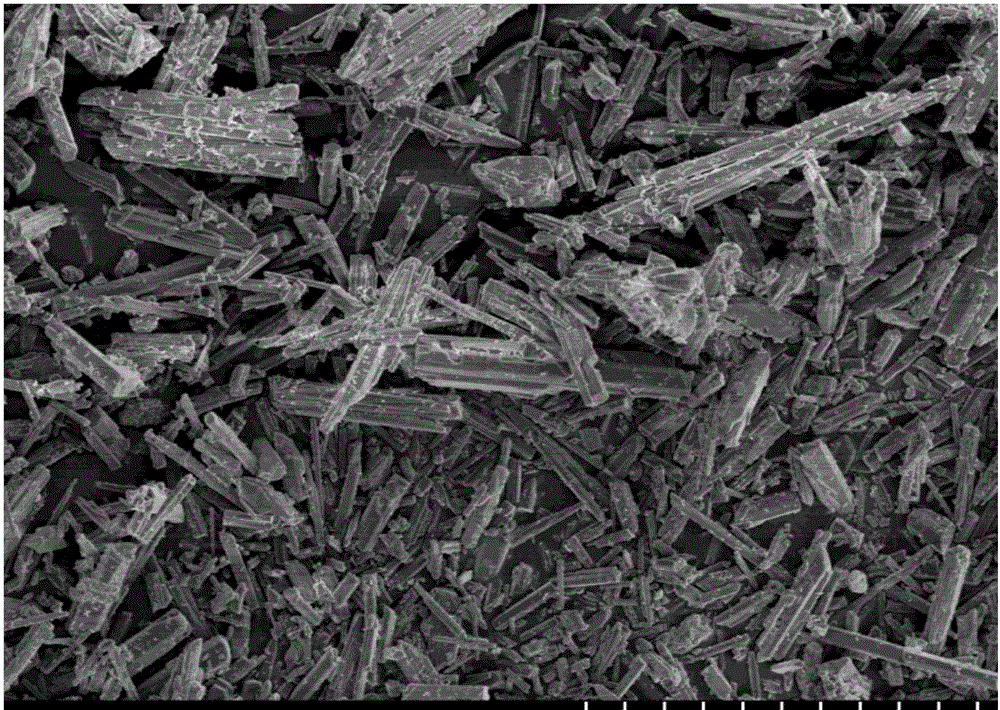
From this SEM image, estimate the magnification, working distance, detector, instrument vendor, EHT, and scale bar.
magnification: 1 K X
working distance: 10 mm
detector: SE(M)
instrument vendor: Hitachi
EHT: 5 kV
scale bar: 50000 nm